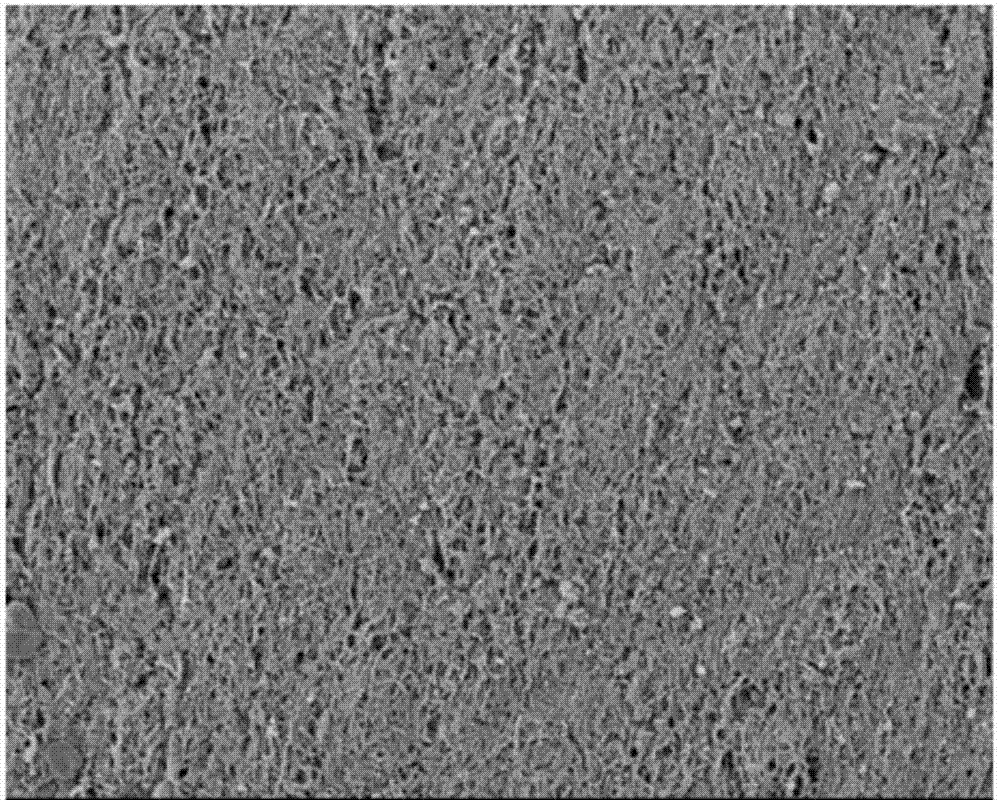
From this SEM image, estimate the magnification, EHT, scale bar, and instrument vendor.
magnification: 1 K X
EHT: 5 kV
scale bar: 20000 nm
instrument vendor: JEOL